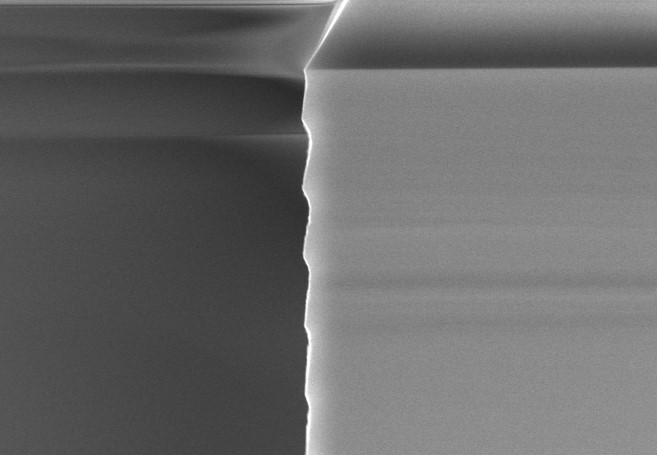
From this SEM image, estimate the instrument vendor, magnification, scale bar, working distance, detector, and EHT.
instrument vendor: Hitachi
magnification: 20 K X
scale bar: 2000 nm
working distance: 4.2 mm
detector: SE(UL)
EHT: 5 kV